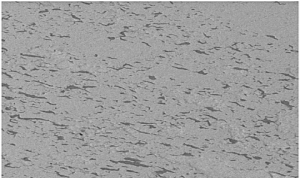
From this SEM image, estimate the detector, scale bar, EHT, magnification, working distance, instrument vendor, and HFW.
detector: BSED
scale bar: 50000 nm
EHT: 20 kV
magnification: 2 K X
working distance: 9.8 mm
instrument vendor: FEI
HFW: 149 µm